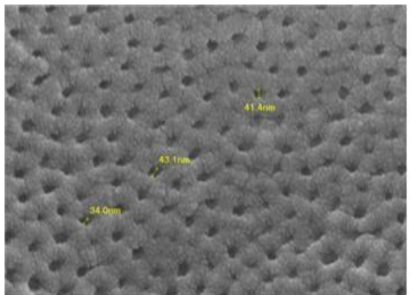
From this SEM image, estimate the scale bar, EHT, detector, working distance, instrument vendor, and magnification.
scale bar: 100 nm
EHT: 10 kV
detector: SEI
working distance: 5.2 mm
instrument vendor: JEOL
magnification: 75 K X